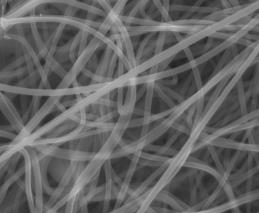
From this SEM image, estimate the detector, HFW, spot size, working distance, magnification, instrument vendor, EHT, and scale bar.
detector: LVD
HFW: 9.95 µm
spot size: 4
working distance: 4.3 mm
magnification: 20 K X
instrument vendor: FEI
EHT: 15 kV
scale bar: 3000 nm